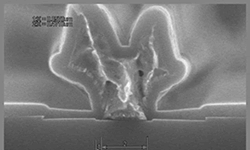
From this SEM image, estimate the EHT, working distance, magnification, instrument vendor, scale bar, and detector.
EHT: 10 kV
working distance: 16.6 mm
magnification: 50 K X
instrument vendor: Hitachi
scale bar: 1000 nm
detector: SE(U)